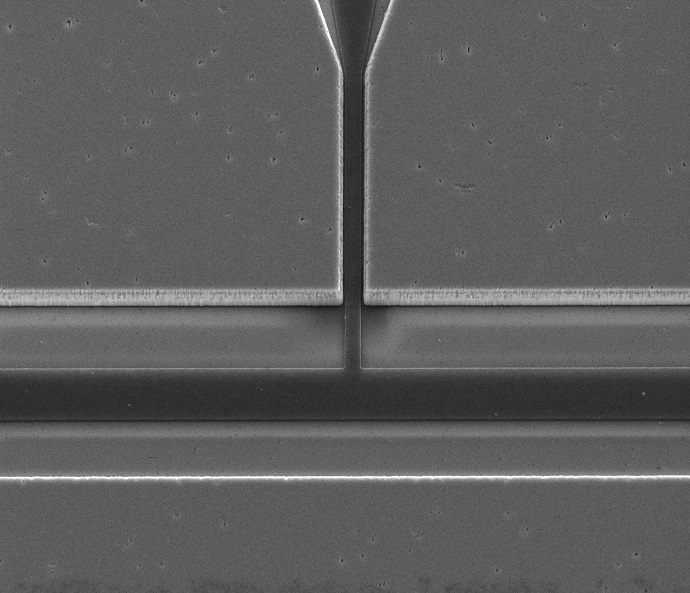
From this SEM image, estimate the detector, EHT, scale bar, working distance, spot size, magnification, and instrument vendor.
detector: ETD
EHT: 10 kV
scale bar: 10000 nm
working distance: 4.2 mm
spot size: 3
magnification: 10 K X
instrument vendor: FEI